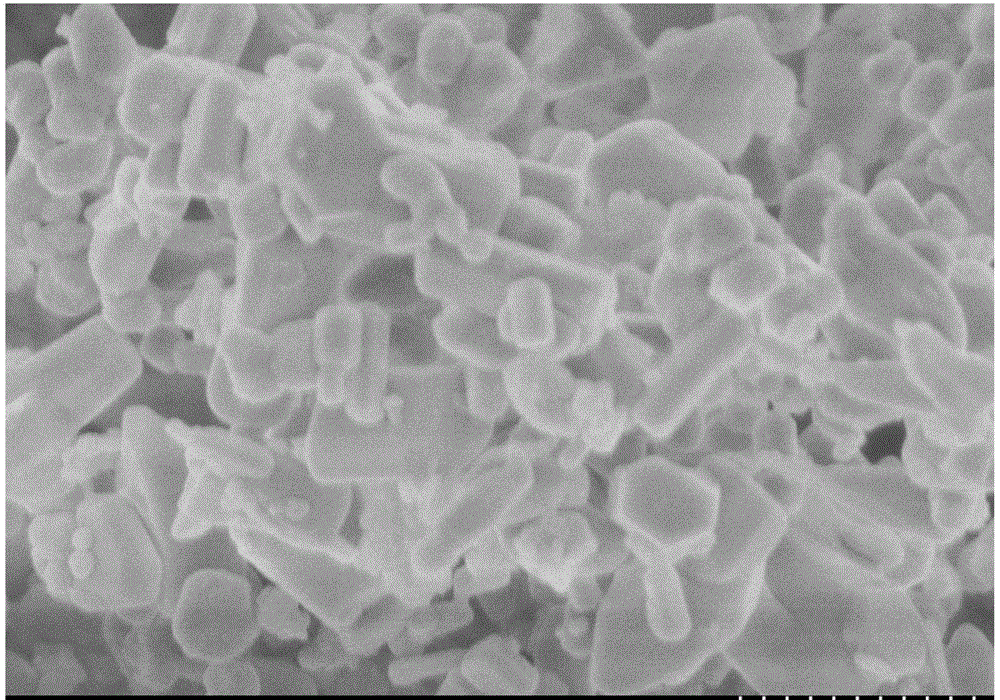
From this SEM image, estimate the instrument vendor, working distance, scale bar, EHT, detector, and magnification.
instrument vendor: Hitachi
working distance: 12.1 mm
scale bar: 1000 nm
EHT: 3 kV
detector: SE(L)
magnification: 30 K X